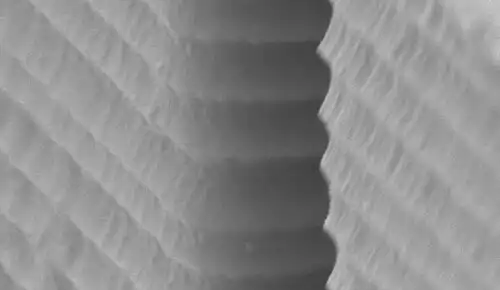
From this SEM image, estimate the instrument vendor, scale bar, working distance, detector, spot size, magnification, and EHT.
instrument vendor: FEI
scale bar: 1000 nm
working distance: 6.3 mm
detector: SE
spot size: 3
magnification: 31.579 K X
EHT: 10 kV